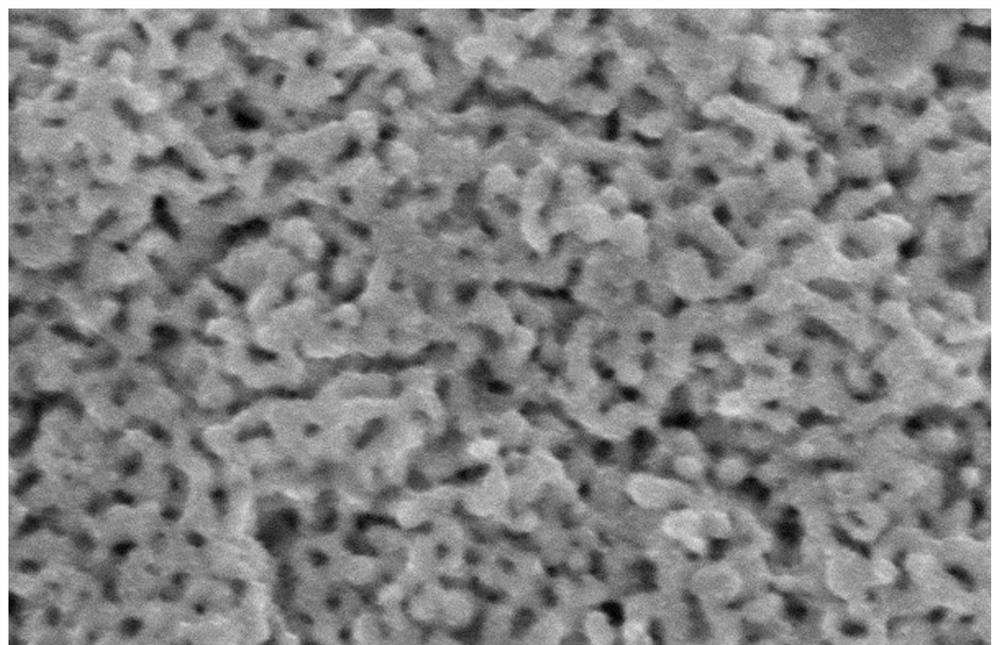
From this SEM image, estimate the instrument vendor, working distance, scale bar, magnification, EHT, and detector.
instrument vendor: Hitachi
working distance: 9.7 mm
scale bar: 1000 nm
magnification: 50 K X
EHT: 10 kV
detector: SE(U)